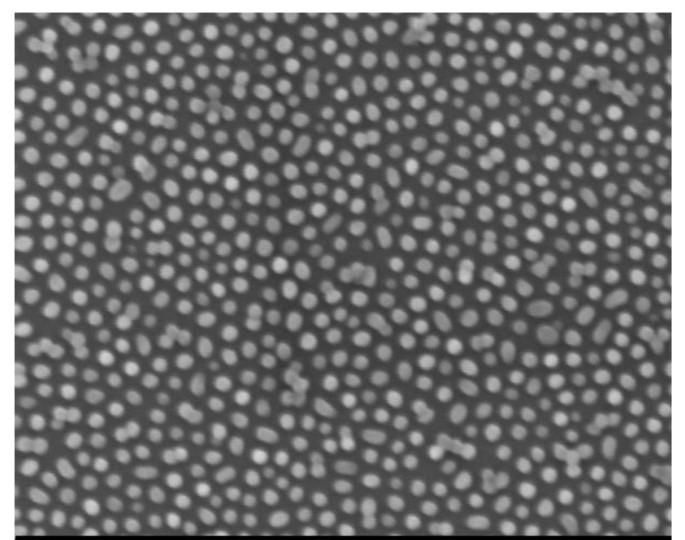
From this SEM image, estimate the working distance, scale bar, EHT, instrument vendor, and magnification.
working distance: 3.3 mm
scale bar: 200 nm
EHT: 1.5 kV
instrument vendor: Zeiss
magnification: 100 K X